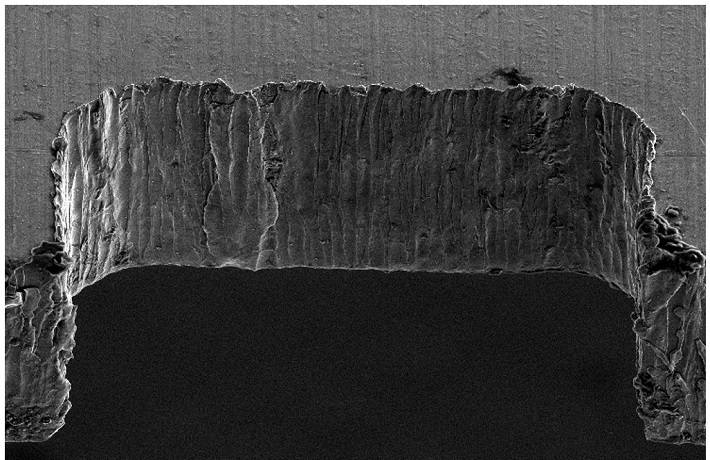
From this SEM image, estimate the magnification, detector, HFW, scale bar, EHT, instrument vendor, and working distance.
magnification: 0.35 K X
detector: ETD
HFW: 363 µm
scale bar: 100000 nm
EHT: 10 kV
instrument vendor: FEI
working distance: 7.8 mm